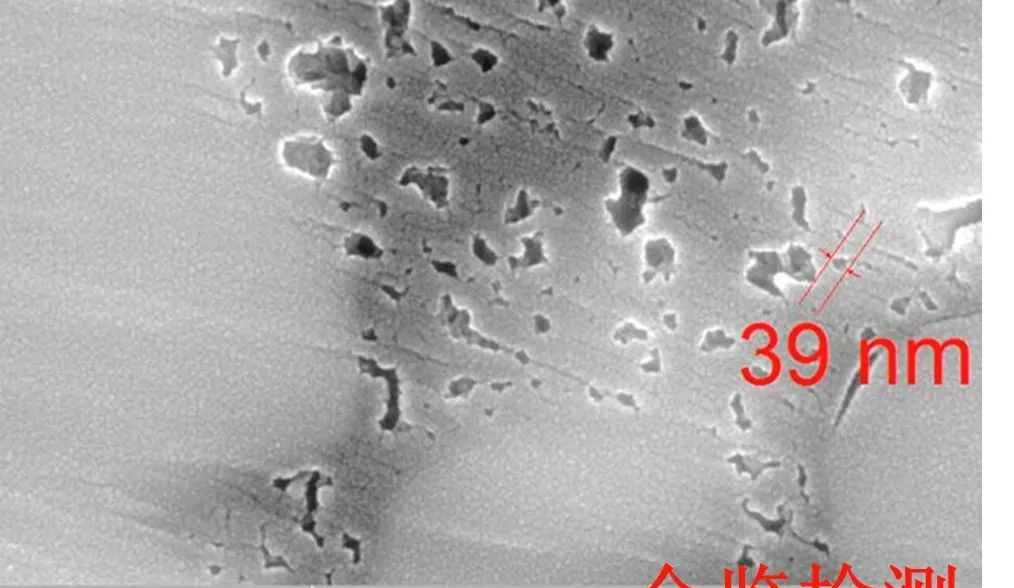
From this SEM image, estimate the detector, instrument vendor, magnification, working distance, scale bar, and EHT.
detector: InBeam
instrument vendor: Tescan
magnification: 100 K X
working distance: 6.03 mm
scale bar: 500 nm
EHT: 20 kV